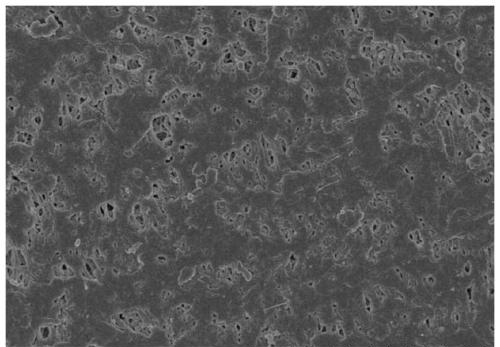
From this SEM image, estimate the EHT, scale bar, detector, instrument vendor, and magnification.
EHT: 4 kV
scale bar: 10000 nm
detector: SE(U)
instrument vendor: Hitachi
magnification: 5 K X